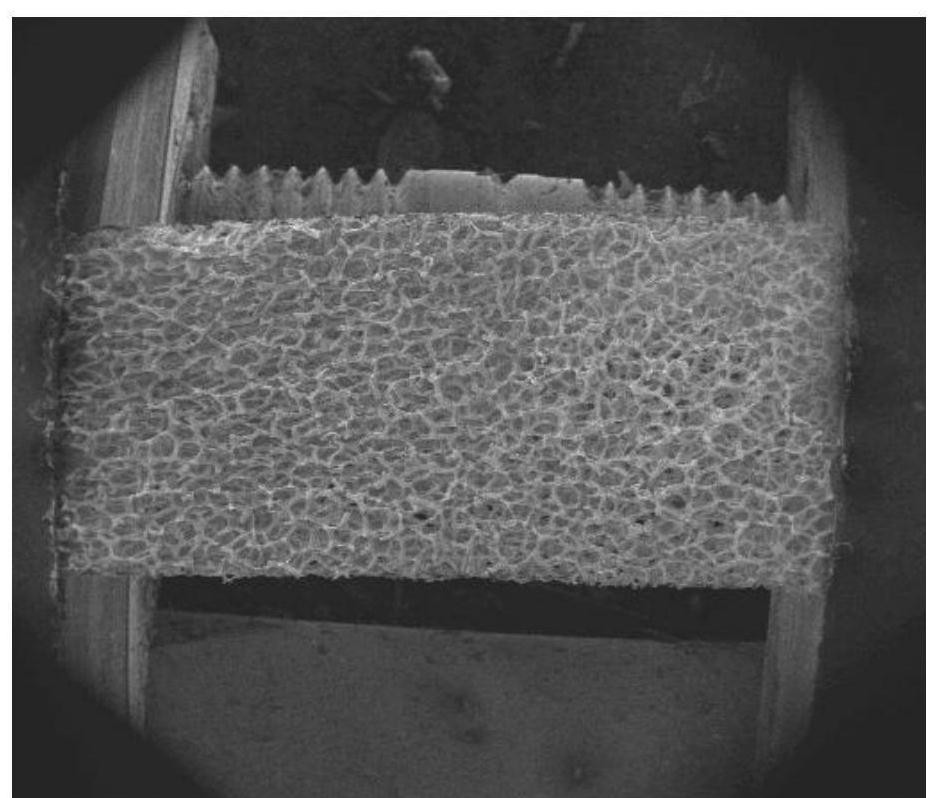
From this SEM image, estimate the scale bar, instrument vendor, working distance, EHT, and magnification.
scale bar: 5000000 nm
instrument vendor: FEI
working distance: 37.1 mm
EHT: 10 kV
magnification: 0.025 K X